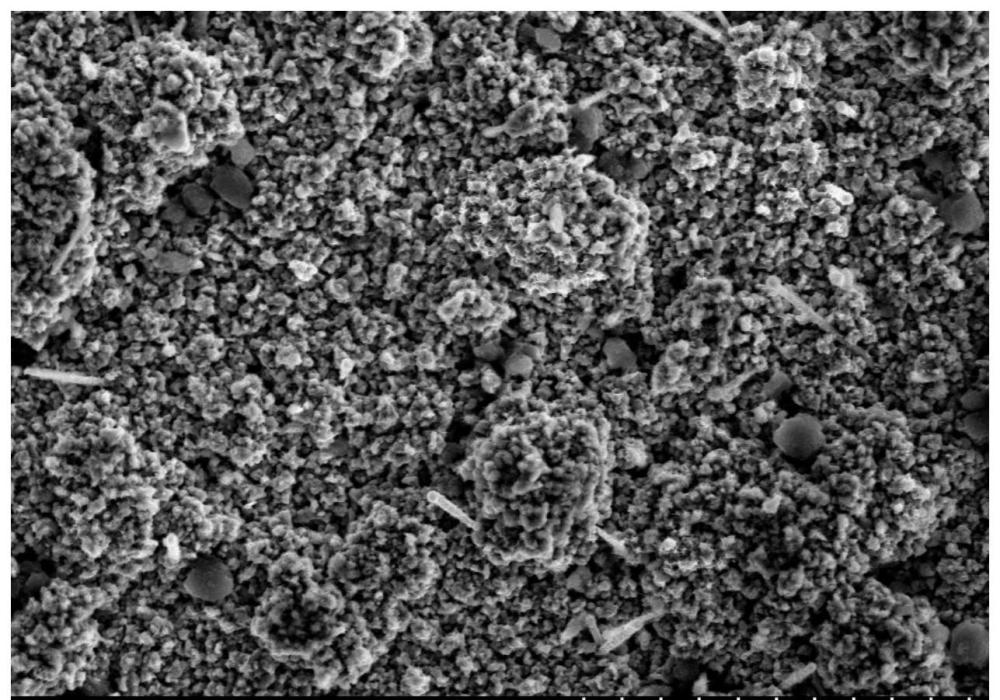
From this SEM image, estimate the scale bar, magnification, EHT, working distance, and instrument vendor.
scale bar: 50000 nm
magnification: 1 K X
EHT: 20 kV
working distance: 14.6 mm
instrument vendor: Hitachi